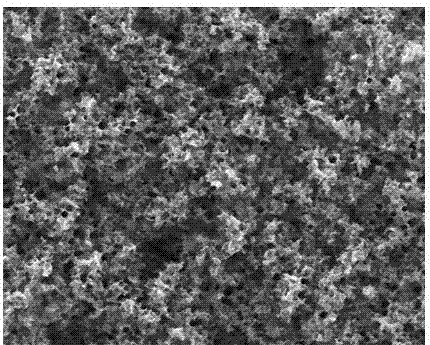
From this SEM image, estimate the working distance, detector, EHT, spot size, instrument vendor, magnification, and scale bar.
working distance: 10.2 mm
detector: ETD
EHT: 30 kV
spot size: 2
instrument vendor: FEI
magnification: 10 K X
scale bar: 10000 nm